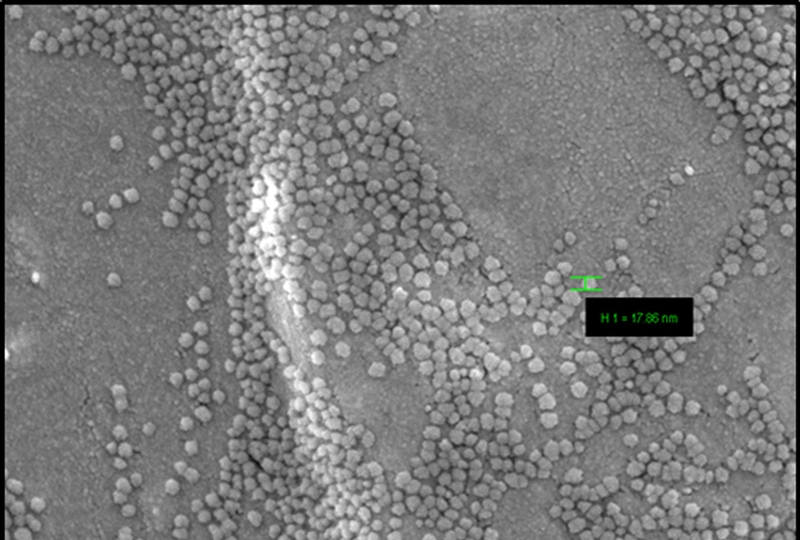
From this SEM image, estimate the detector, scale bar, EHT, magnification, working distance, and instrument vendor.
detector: InLens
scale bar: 100 nm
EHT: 3 kV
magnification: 100 K X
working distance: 4.8 mm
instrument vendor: Zeiss